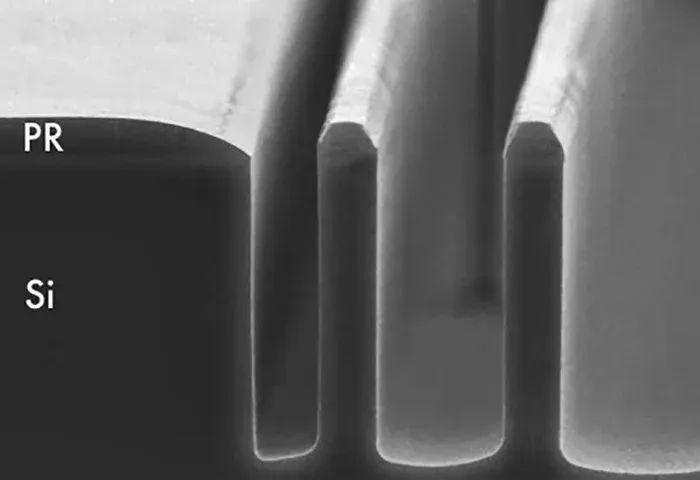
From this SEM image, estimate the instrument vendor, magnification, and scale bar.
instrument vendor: other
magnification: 1 K X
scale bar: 10000 nm